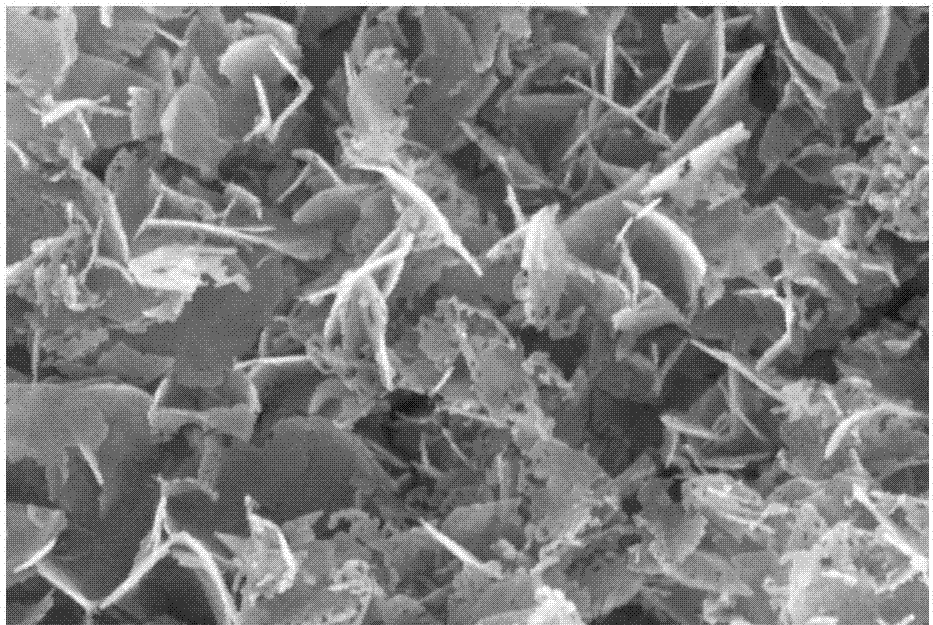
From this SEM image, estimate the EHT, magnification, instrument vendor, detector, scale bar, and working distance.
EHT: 20 kV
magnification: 50 K X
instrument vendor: Zeiss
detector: InLens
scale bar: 100 nm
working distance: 6.2 mm